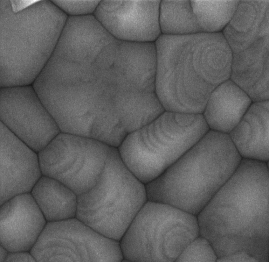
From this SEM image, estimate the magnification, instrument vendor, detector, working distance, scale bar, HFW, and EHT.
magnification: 101 K X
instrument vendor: Tescan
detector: InBeam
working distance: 8.82 mm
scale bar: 500 nm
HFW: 2.06 µm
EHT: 10 kV